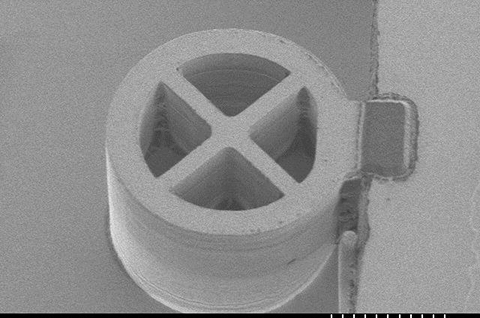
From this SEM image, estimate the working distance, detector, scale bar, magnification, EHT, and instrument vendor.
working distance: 10.1 mm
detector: SE(M)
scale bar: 10000 nm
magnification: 3 K X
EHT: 1 kV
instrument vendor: Hitachi